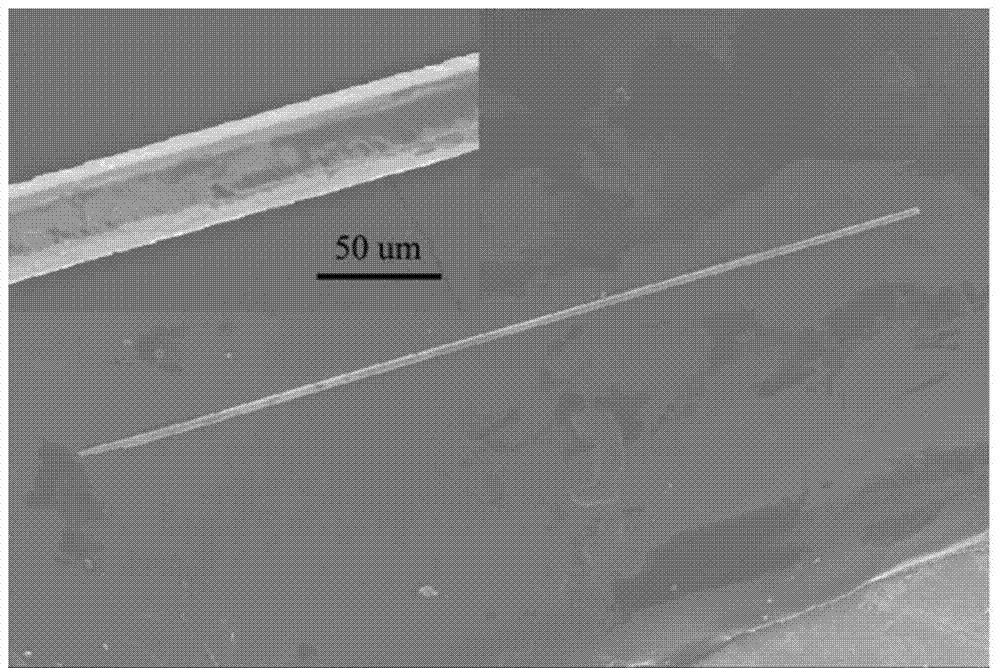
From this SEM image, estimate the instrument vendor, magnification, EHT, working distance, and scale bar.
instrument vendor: Hitachi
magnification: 0.04 K X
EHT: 20 kV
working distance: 11.2 mm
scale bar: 1000000 nm